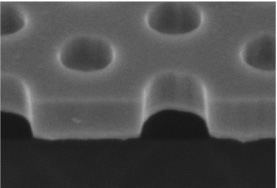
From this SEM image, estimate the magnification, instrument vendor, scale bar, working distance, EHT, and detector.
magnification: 120 K X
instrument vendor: Hitachi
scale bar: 400 nm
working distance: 11.1 mm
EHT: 2 kV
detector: SE(M)